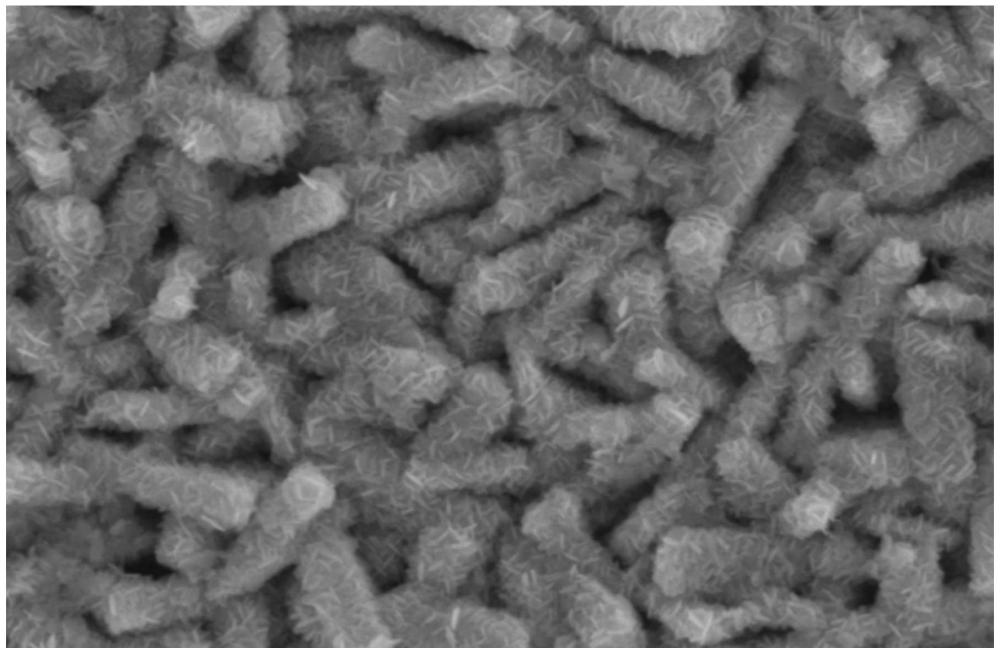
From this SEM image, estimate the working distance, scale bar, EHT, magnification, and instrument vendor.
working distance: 10 mm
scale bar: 3000 nm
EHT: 10 kV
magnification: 18 K X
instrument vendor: Hitachi